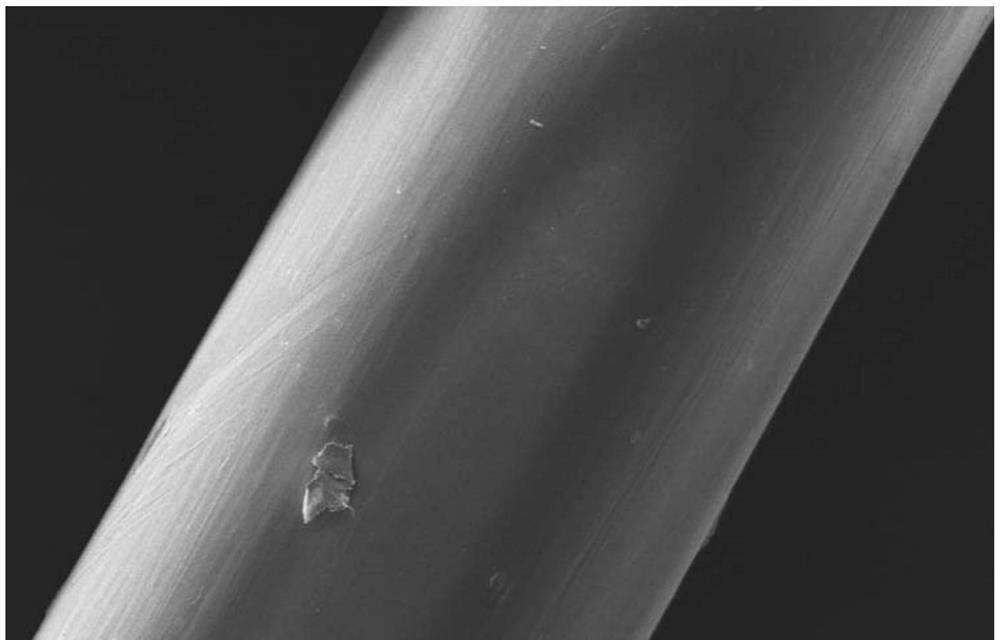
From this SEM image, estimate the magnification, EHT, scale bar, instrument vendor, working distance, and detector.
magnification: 0.2 K X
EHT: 5 kV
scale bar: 200000 nm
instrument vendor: Hitachi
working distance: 10.6 mm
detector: SE(M)